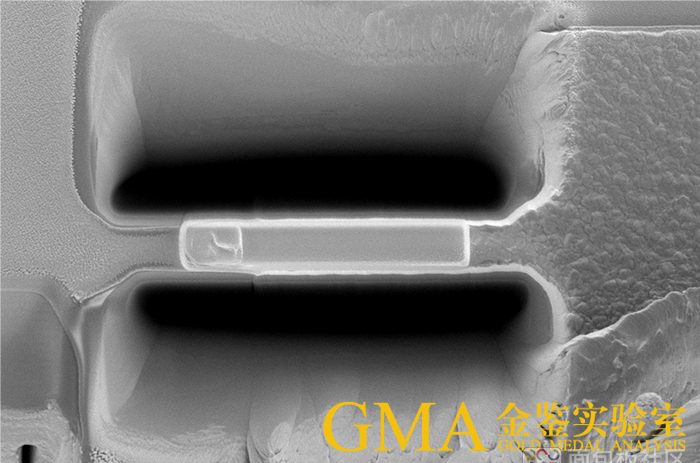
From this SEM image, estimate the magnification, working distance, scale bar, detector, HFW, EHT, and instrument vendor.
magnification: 3.5 K X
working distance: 7 mm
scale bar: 10000 nm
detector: ETD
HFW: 36.3 µm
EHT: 3 kV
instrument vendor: FEI